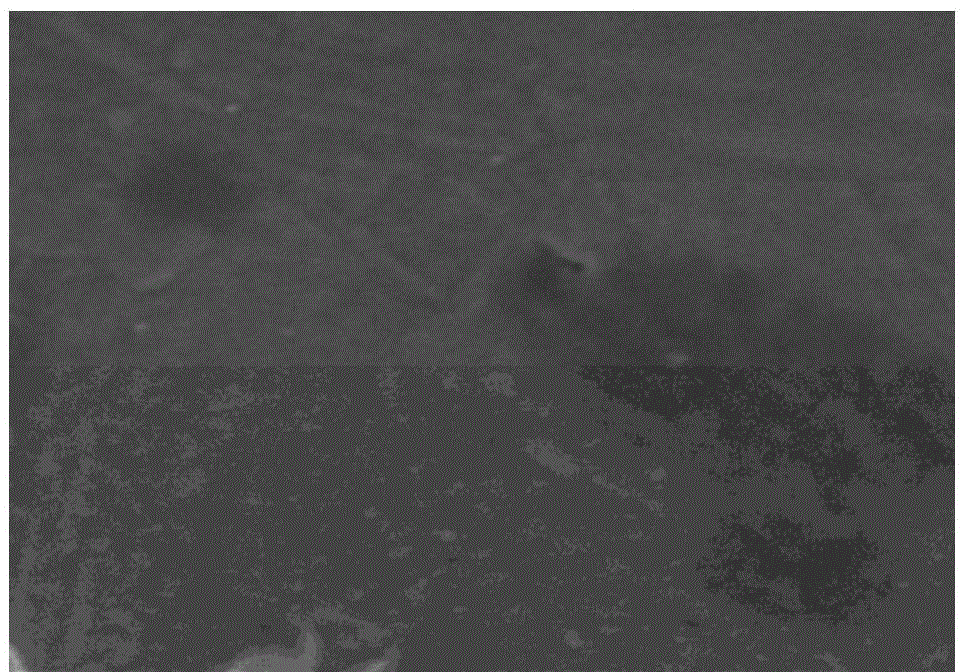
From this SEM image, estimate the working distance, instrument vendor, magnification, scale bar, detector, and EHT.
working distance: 8.1 mm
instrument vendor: Hitachi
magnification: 50 K X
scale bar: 1000 nm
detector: SE(M)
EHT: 3 kV